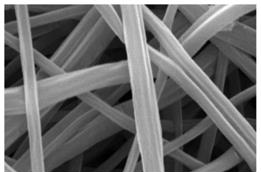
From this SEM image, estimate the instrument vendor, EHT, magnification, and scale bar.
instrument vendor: JEOL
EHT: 15 kV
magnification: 10 K X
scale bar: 1000 nm